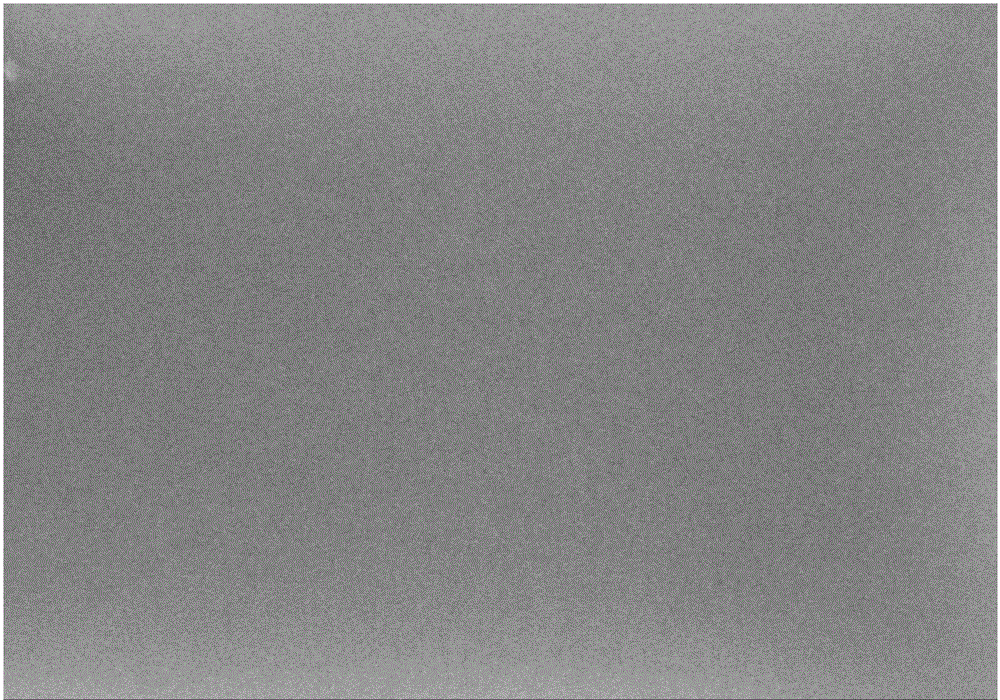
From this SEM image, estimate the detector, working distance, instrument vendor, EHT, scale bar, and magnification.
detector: SE(M)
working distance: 11.8 mm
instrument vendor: Hitachi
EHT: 5 kV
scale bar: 1000 nm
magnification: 50 K X